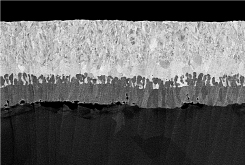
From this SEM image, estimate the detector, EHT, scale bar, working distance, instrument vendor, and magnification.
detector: PDBSE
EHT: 5 kV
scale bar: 5000 nm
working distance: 8.8 mm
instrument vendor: Hitachi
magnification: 10 K X